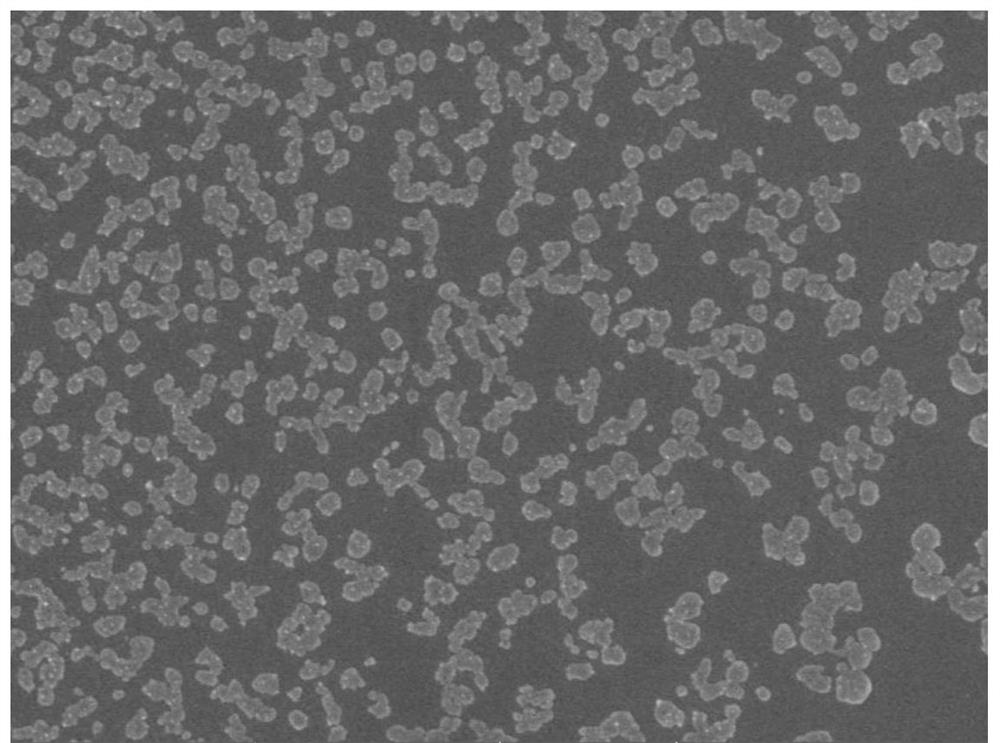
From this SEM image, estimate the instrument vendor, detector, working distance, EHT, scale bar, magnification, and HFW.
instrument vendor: Tescan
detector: In-Beam SE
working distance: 3.98 mm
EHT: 5 kV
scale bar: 1000 nm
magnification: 50 K X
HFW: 5.54 µm